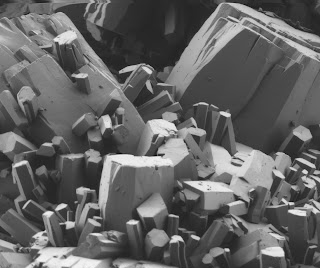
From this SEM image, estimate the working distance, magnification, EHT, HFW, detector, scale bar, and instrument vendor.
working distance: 4.2 mm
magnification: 2 K X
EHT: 1 kV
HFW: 75.4 µm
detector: BSED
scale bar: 30000 nm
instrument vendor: FEI